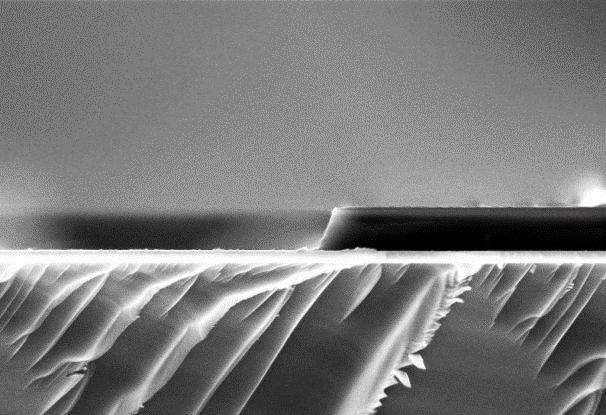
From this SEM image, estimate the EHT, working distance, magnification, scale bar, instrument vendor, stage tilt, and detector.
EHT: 5 kV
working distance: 4.7 mm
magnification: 50 K X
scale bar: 200 nm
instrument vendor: Zeiss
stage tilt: -1.5°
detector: InLens